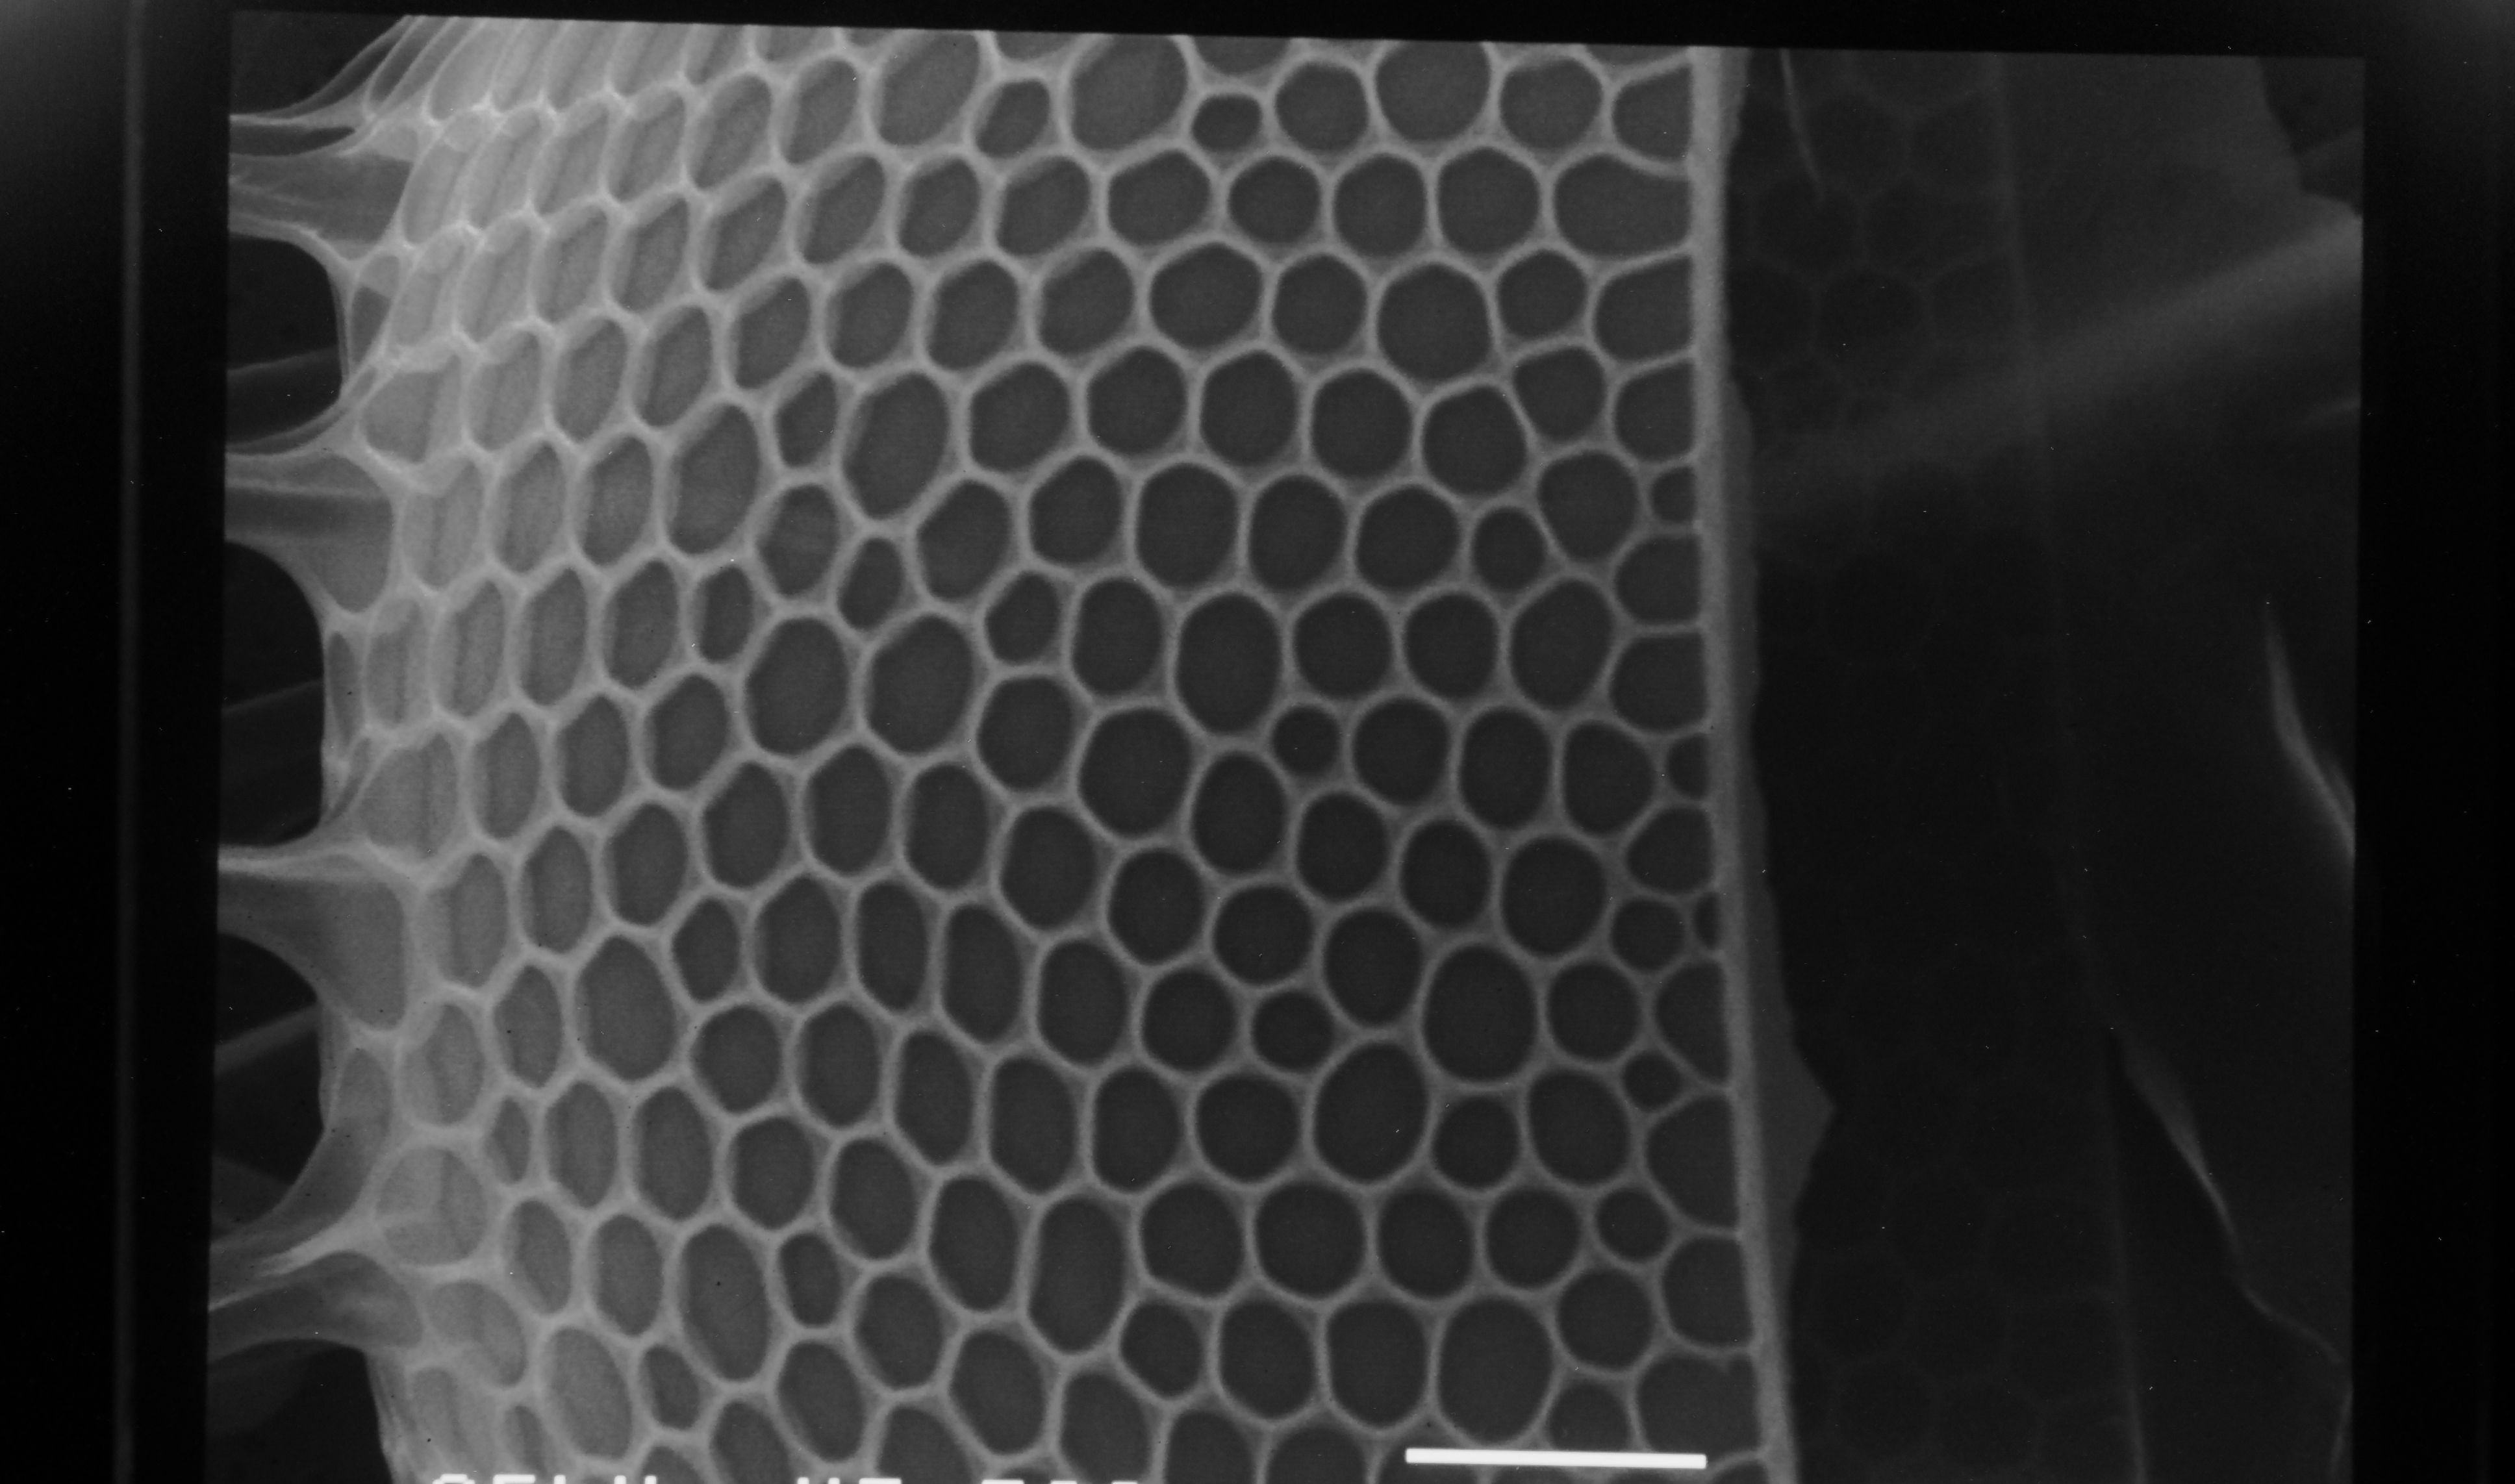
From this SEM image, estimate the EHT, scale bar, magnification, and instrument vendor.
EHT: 25 kV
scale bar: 5000 nm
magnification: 3.5 K X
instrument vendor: JEOL